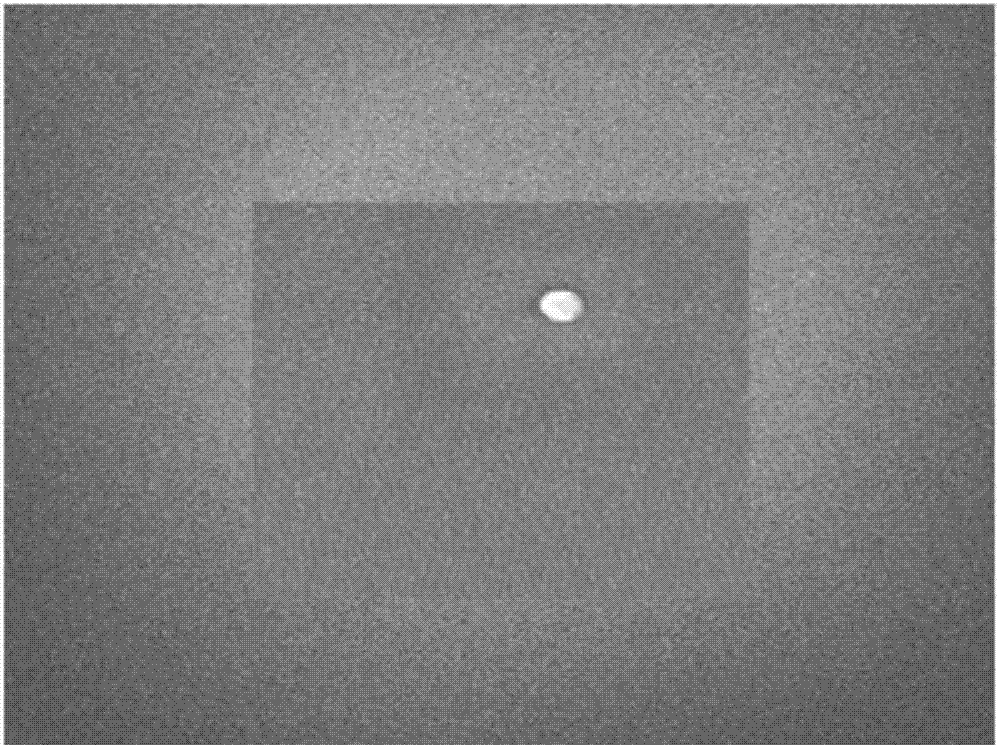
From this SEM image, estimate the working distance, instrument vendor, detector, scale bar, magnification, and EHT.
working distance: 5.7 mm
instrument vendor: JEOL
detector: SEI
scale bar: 100 nm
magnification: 70 K X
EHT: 3 kV